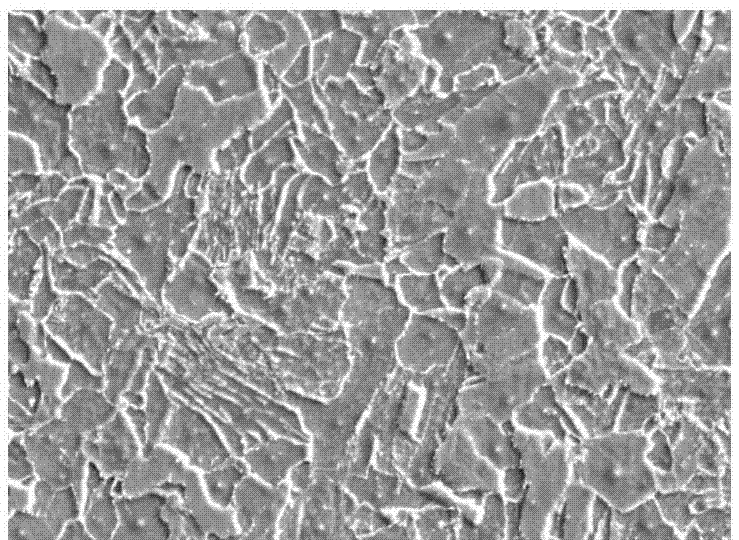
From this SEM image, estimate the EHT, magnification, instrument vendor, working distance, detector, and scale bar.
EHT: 20 kV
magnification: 1.5 K X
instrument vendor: JEOL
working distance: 10.8 mm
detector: SEI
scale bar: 10000 nm